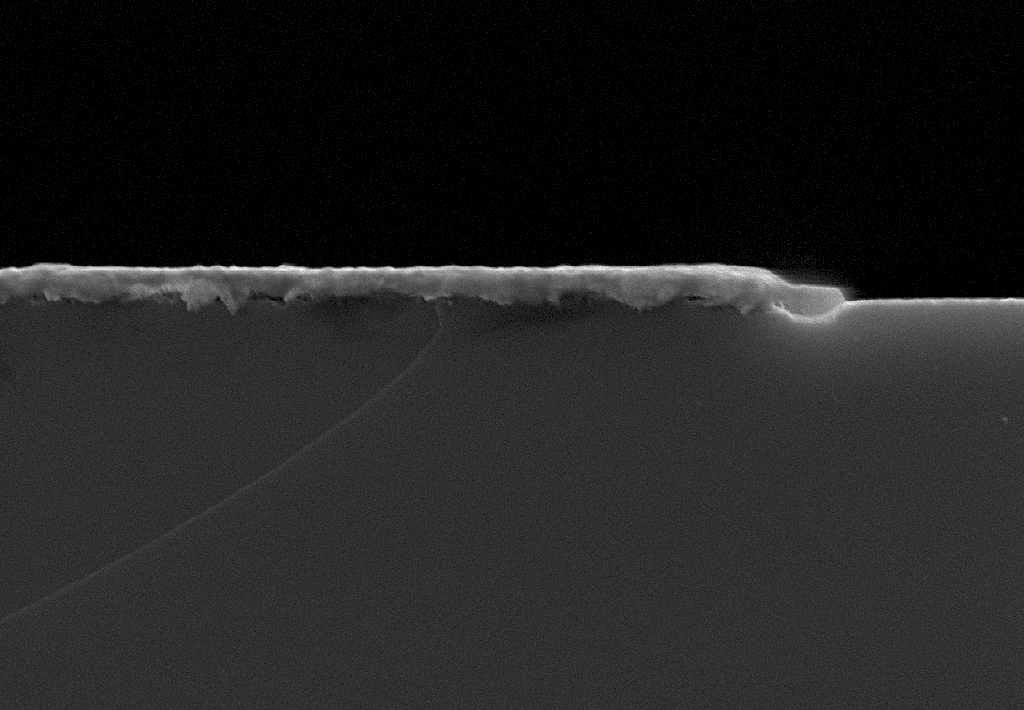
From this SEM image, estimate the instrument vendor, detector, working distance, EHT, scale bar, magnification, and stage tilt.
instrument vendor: Zeiss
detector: InLens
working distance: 3.1 mm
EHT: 7 kV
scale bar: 200 nm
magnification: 100 K X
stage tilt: -1.5°